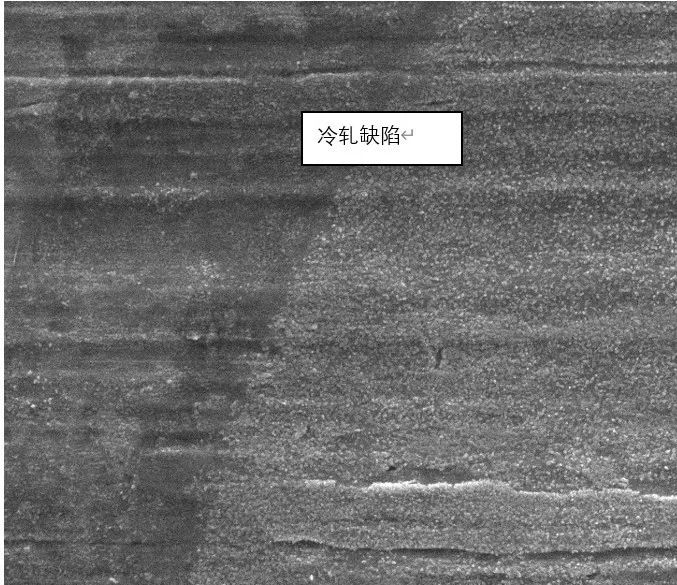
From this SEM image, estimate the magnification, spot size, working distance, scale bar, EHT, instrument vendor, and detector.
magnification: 2 K X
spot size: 4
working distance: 11 mm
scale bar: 20000 nm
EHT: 20 kV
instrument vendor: FEI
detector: ETD